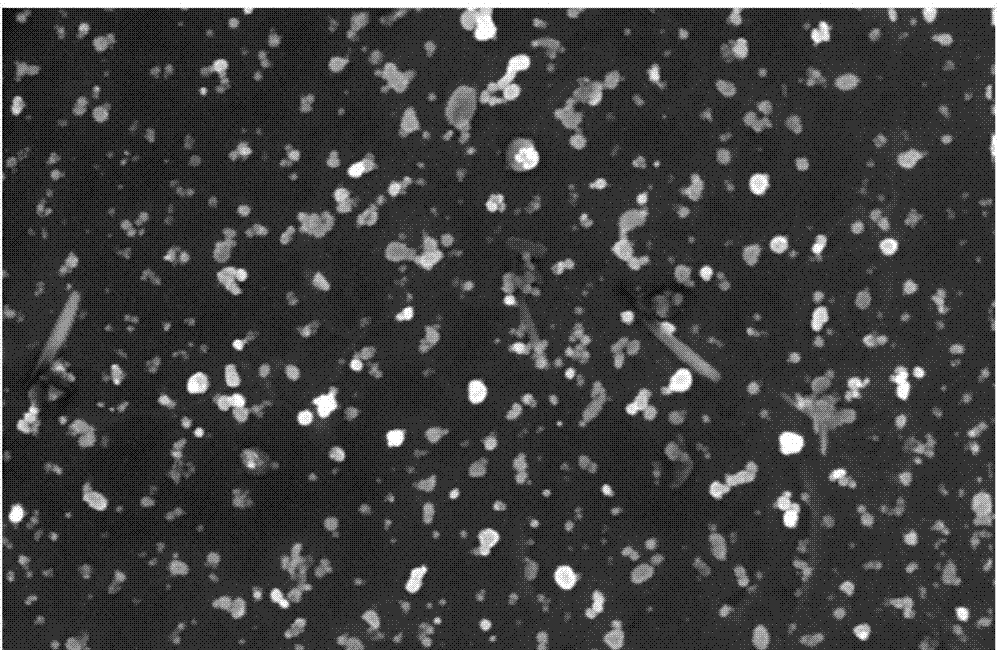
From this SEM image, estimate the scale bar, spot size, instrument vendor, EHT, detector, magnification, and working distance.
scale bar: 1000 nm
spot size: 4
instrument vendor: FEI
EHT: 5 kV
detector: TLD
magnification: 50 K X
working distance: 4.9 mm